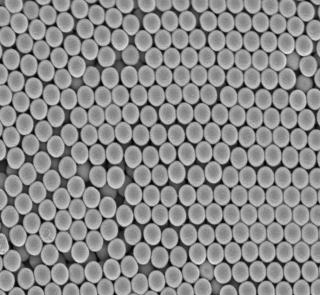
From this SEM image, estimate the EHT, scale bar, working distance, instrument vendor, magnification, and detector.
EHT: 1 kV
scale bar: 100 nm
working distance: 4 mm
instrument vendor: JEOL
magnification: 30 K X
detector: UED